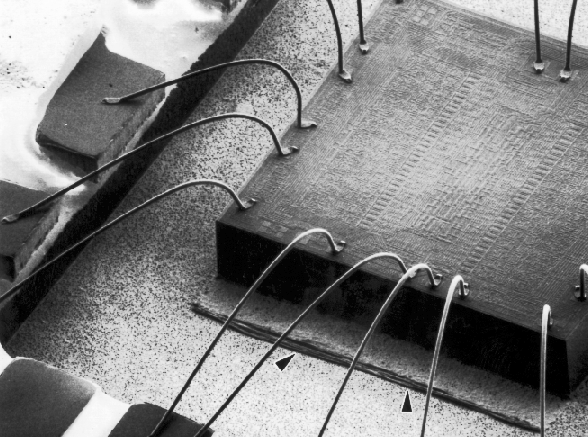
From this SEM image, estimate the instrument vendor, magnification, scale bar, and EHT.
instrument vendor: JEOL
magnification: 0.0276 K X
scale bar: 362000 nm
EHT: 15 kV